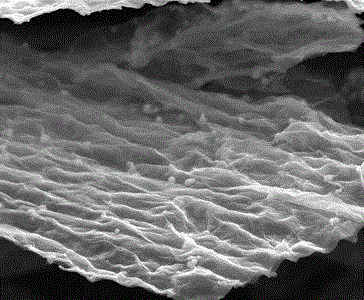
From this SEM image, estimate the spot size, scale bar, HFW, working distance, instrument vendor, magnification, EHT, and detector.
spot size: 2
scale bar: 4000 nm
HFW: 14.9 µm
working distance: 5.8 mm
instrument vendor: FEI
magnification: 10 K X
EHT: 10 kV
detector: ETD